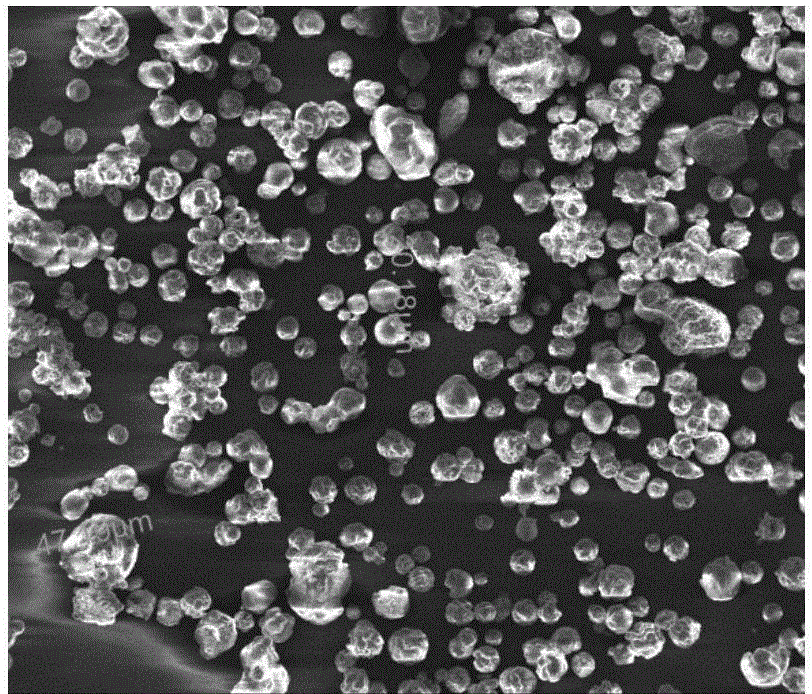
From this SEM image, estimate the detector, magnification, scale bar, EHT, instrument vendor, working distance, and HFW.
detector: ETD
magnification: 0.5 K X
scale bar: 100000 nm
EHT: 10 kV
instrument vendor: FEI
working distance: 5.1 mm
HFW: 480 µm